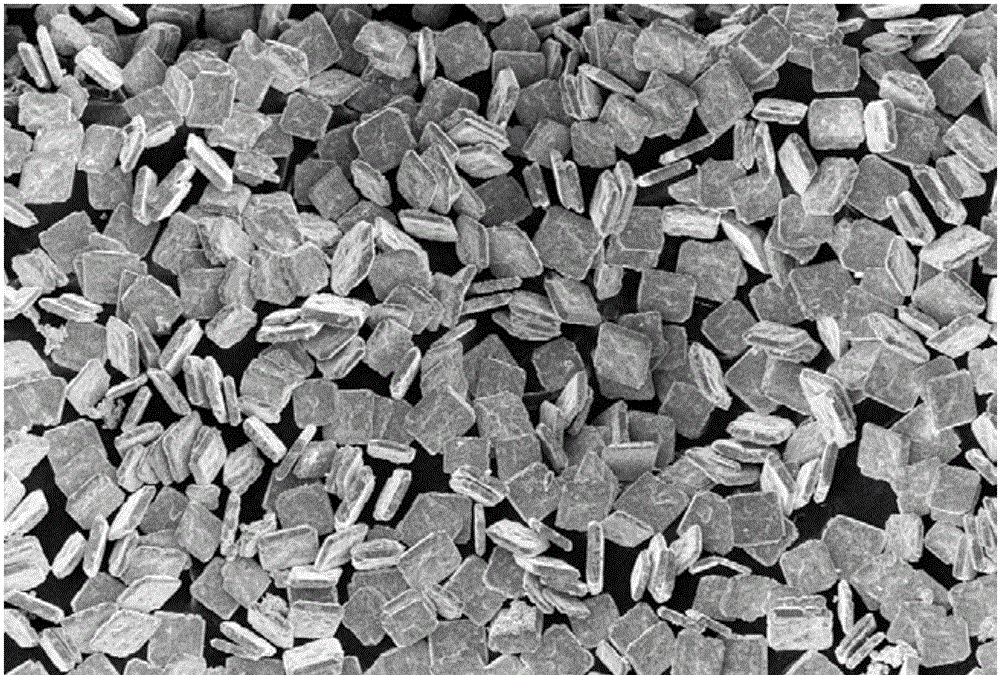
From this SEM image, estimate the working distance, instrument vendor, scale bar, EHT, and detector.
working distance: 8.6 mm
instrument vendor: Zeiss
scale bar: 100000 nm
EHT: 10 kV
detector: InLens